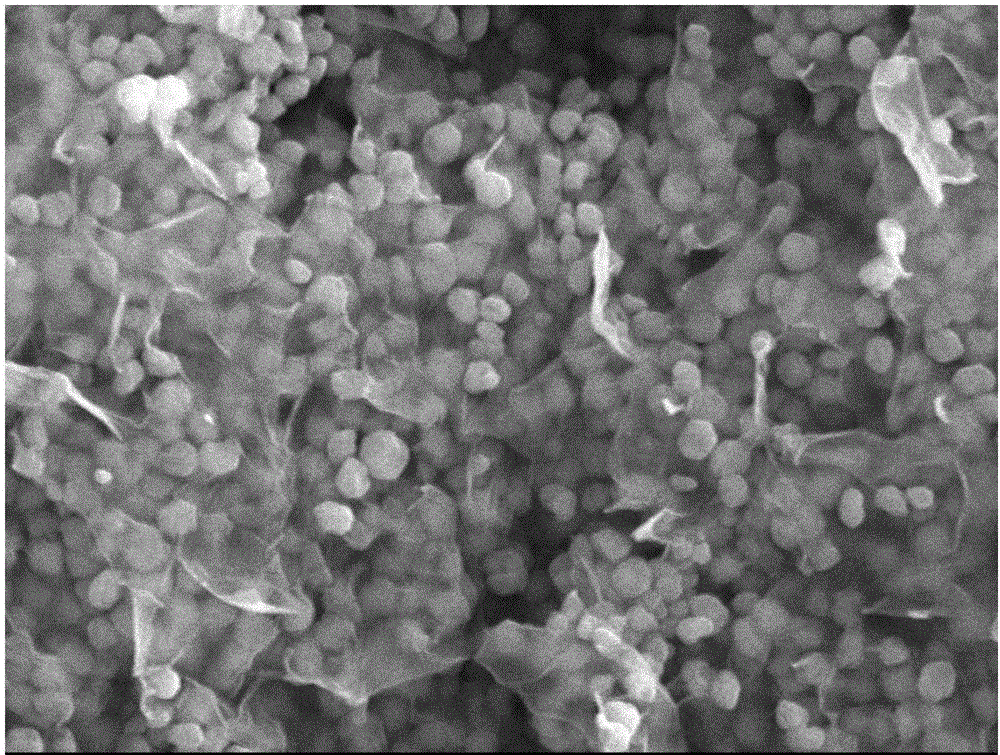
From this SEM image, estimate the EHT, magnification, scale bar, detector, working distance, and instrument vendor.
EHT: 15 kV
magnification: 20 K X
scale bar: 1000 nm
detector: SEI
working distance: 12.1 mm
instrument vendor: JEOL